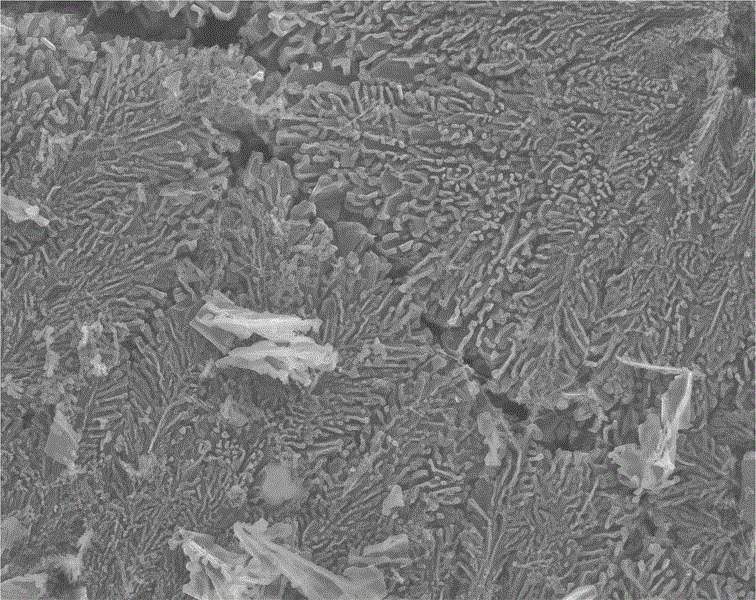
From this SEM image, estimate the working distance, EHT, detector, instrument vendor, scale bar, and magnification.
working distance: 6.2 mm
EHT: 10 kV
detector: SEI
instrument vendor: JEOL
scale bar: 1000 nm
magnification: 5 K X